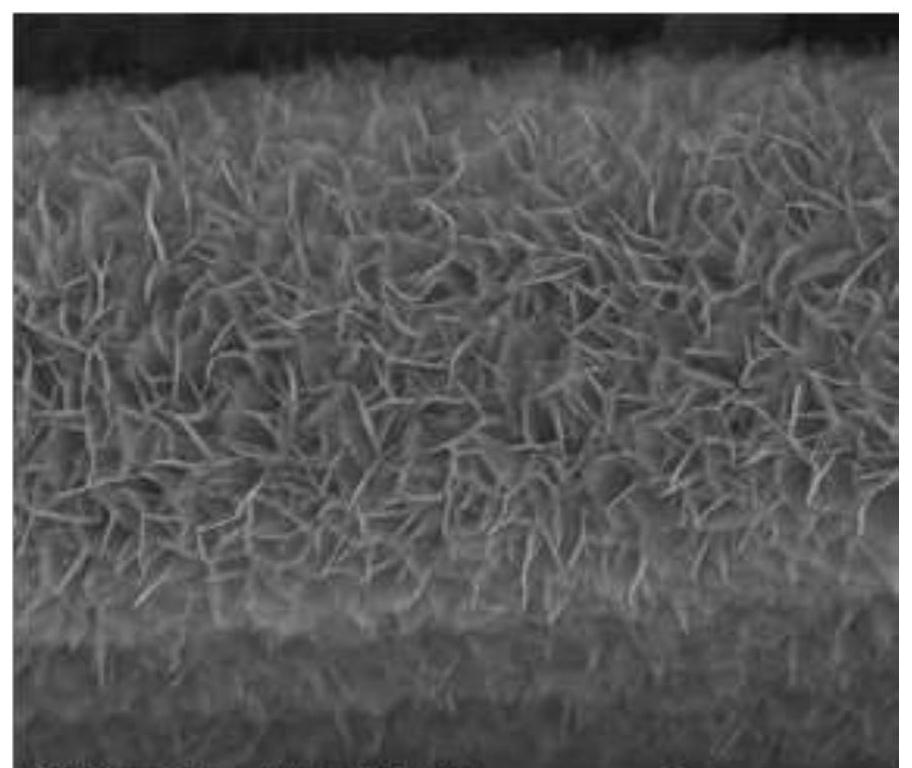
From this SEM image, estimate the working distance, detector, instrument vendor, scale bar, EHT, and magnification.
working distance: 10.6 mm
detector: ETD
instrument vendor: FEI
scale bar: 5000 nm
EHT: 25 kV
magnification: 24 K X